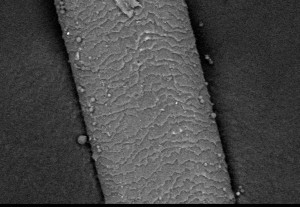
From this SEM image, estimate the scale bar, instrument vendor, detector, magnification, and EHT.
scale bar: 50000 nm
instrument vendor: JEOL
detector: BES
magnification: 0.5 K X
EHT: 15 kV